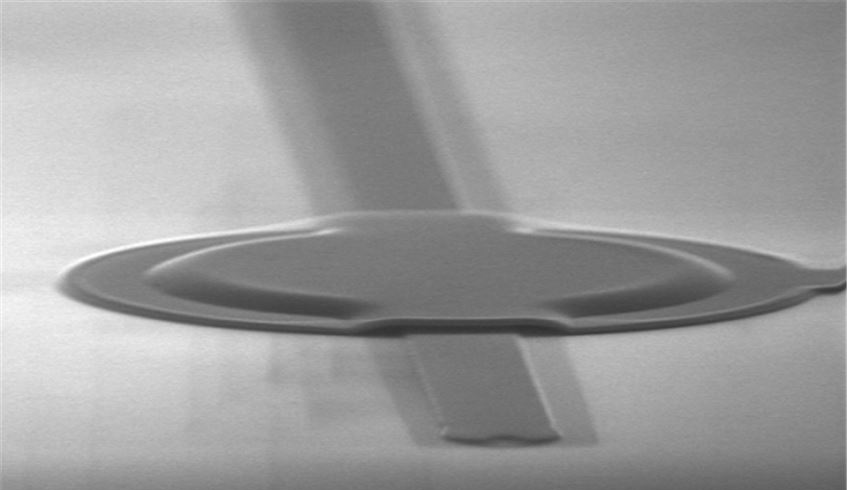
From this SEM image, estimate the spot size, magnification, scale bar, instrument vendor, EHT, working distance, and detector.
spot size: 3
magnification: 6.708 K X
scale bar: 5000 nm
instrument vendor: FEI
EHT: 30 kV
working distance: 6.3 mm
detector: TLD